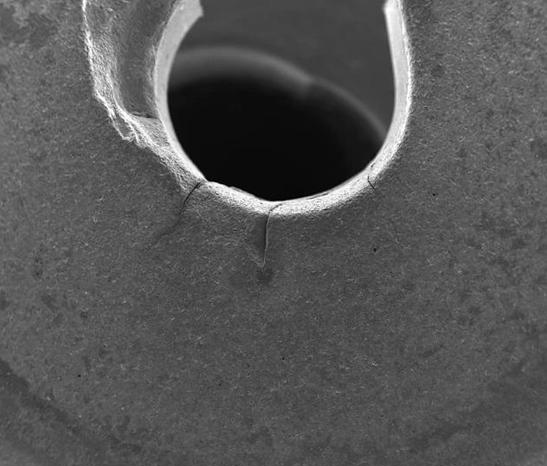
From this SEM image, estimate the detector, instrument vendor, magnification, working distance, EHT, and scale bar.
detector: ETD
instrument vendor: FEI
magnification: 0.07 K X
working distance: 10 mm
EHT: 25 kV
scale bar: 1000000 nm